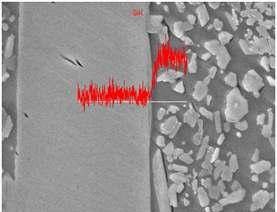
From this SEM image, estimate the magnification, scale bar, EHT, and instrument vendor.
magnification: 5 K X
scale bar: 5000 nm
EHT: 15 kV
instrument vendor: JEOL